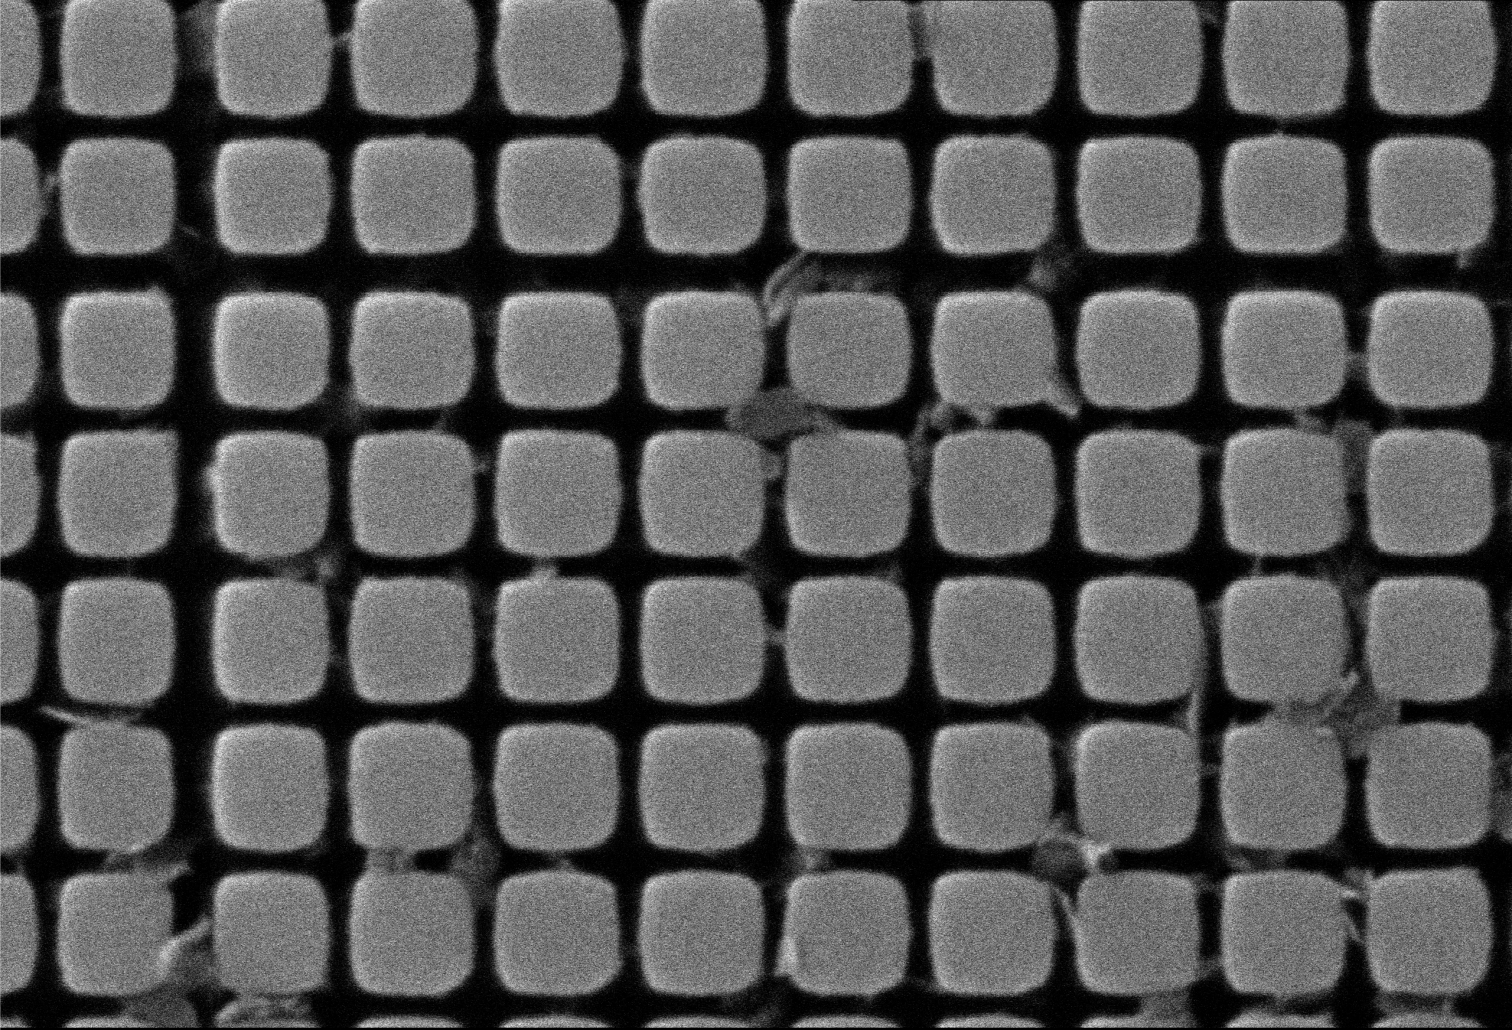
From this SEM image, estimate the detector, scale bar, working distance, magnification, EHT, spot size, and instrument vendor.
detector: SEI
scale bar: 1000 nm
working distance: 10 mm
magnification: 12 K X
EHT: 25 kV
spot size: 45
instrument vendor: JEOL